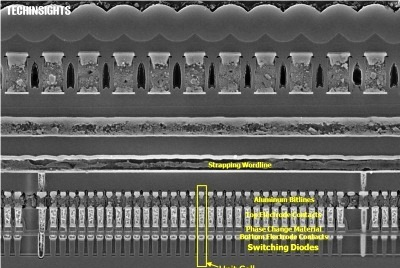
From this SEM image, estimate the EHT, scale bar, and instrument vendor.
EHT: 5 kV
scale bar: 1000 nm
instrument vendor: Zeiss